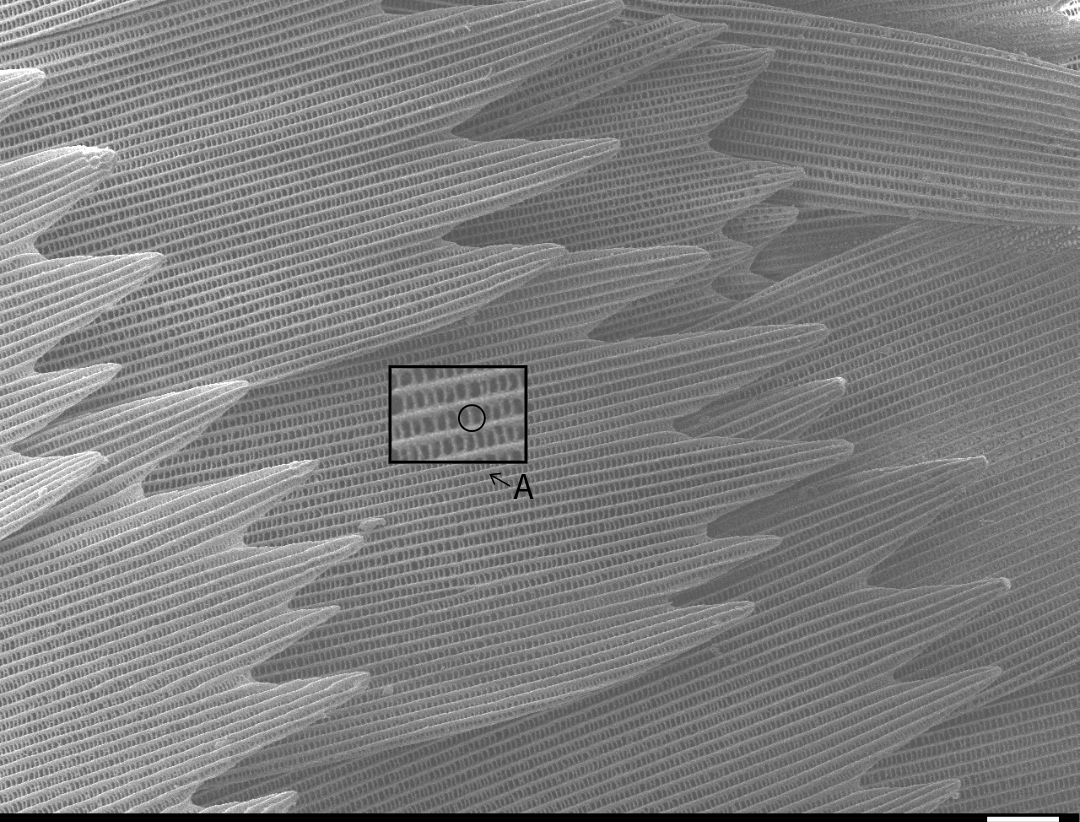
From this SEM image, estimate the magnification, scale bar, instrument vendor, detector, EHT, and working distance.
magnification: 0.8 K X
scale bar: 10000 nm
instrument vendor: JEOL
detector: SEI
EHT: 5 kV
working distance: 13.2 mm